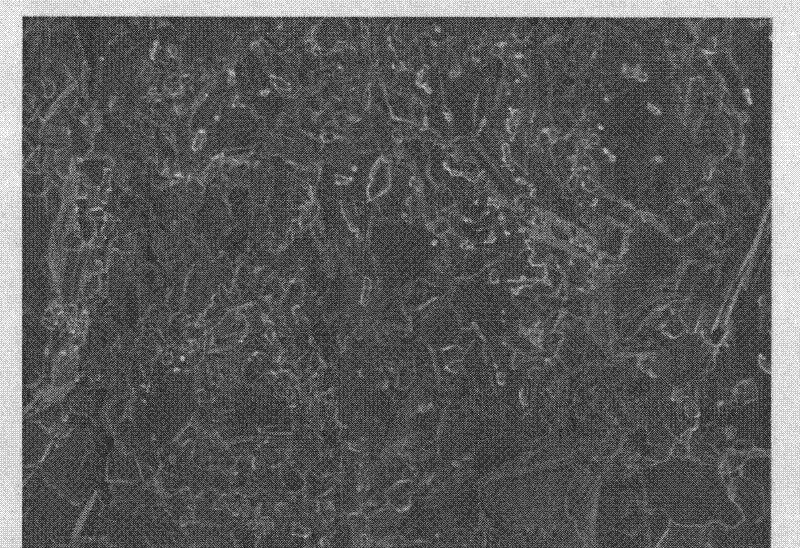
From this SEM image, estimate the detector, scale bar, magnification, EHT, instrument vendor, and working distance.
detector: SEI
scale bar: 10000 nm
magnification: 2 K X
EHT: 5 kV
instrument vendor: JEOL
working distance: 6 mm